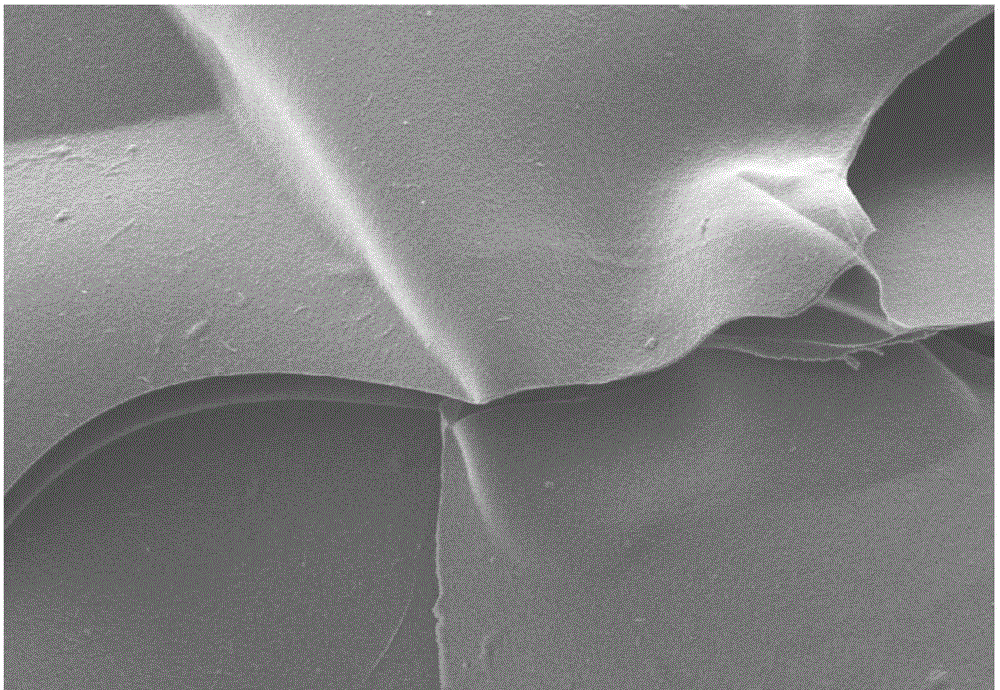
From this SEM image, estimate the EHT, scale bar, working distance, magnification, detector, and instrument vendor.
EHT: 10 kV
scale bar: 200000 nm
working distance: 8.2 mm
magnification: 0.2 K X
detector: LM(UL)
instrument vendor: Hitachi